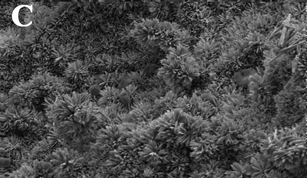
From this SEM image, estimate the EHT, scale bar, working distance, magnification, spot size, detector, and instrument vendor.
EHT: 5 kV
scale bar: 5000 nm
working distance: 8.2 mm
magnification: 10 K X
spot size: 4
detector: SE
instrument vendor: FEI